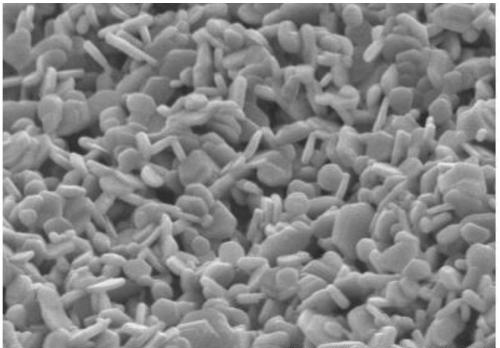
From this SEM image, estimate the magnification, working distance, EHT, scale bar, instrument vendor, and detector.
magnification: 5 K X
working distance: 15.5 mm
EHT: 15 kV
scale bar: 10000 nm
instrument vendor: Hitachi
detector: SE